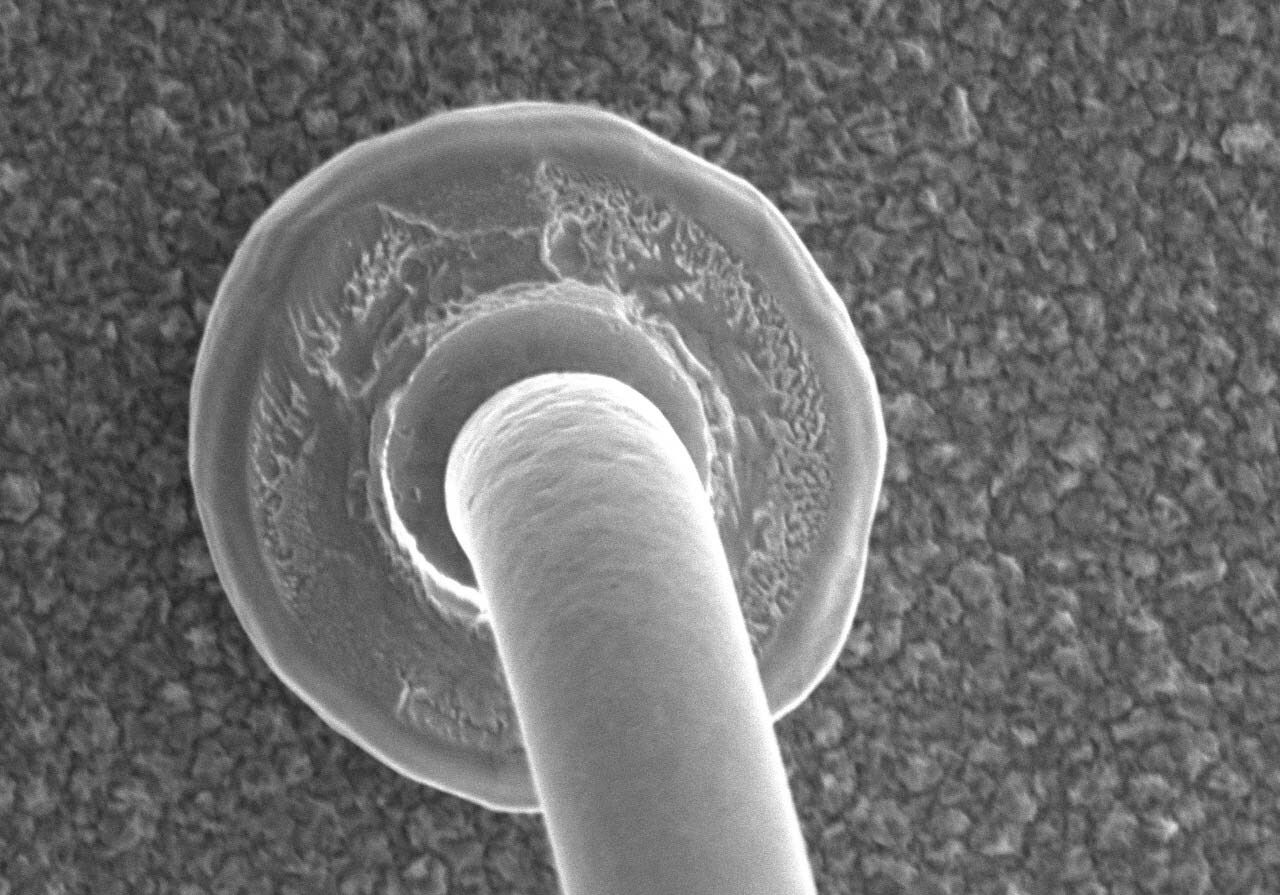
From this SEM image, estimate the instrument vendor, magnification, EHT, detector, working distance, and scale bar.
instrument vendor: Hitachi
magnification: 1.3 K X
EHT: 25 kV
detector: SE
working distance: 11 mm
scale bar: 40000 nm